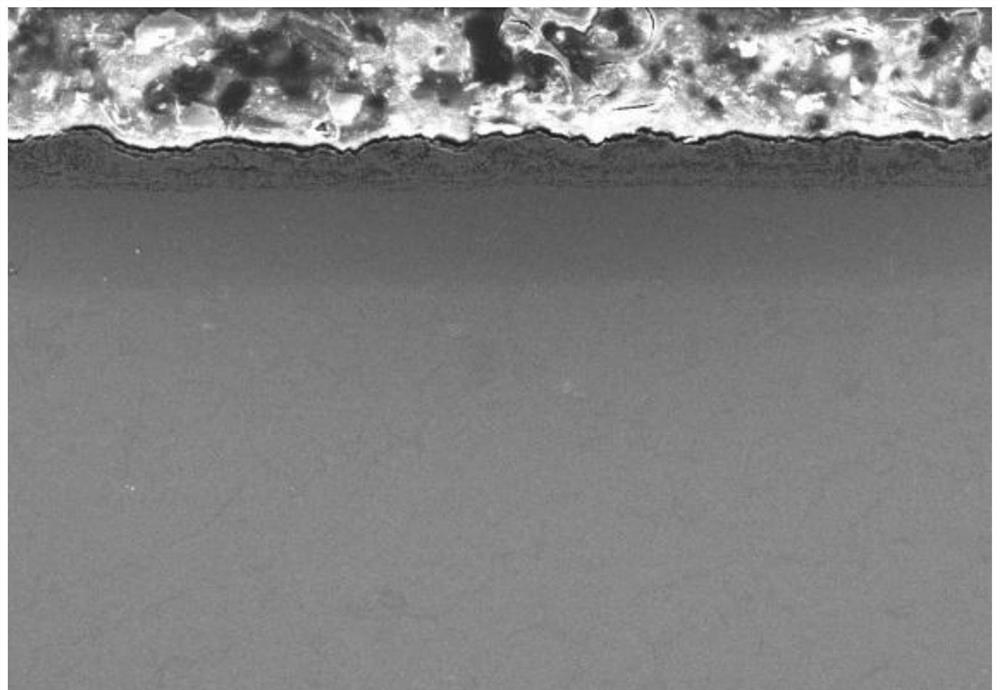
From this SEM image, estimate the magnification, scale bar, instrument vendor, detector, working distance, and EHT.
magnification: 0.2 K X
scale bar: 200000 nm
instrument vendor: Hitachi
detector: SE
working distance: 9.5 mm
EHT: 15 kV